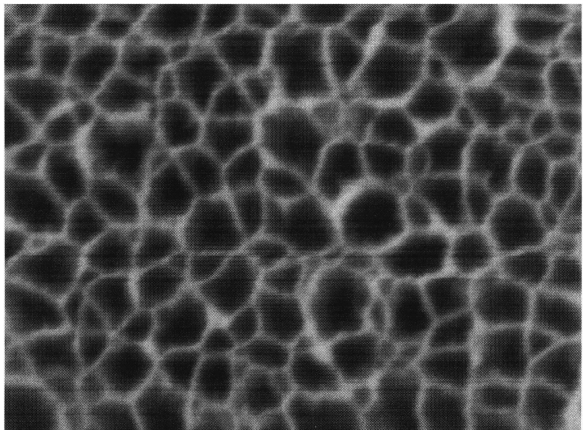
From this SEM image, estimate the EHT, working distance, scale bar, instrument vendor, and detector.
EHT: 5 kV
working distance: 9 mm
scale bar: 100 nm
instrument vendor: JEOL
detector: SEI